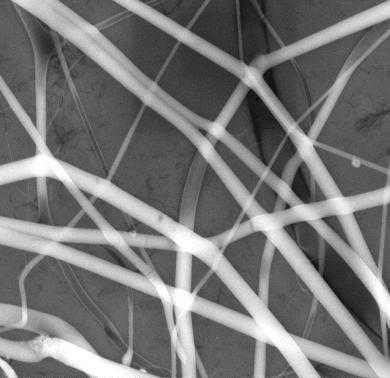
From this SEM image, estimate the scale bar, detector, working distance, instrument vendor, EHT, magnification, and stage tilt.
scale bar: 5000 nm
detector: Helix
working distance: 5.7 mm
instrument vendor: FEI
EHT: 10 kV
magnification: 20 K X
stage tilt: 0°